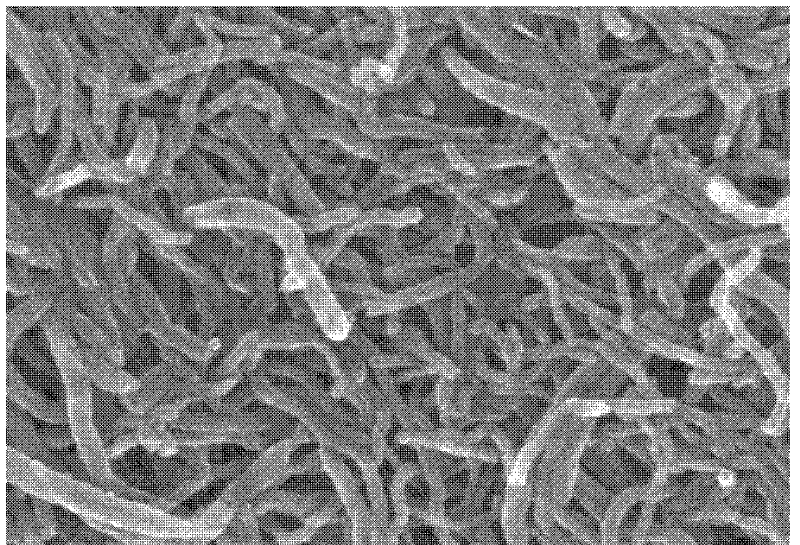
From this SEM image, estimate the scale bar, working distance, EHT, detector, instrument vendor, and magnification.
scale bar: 500 nm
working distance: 8.1 mm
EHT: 20 kV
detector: SE(M)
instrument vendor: Hitachi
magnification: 100 K X